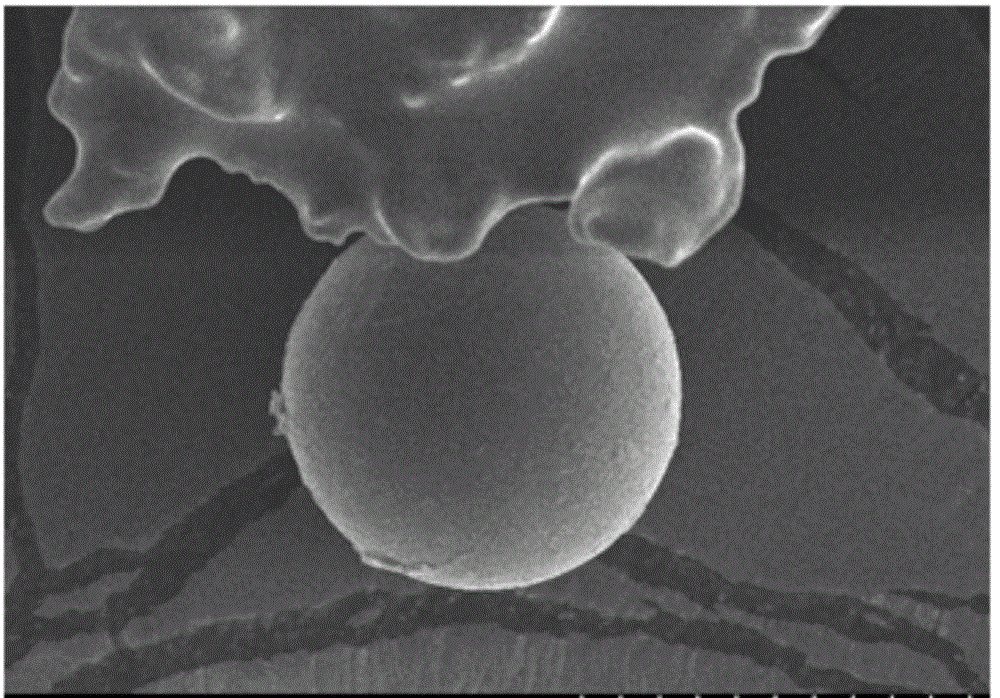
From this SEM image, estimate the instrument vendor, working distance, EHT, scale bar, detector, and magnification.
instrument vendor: Hitachi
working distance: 12 mm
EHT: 10 kV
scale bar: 50000 nm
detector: SE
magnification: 1 K X